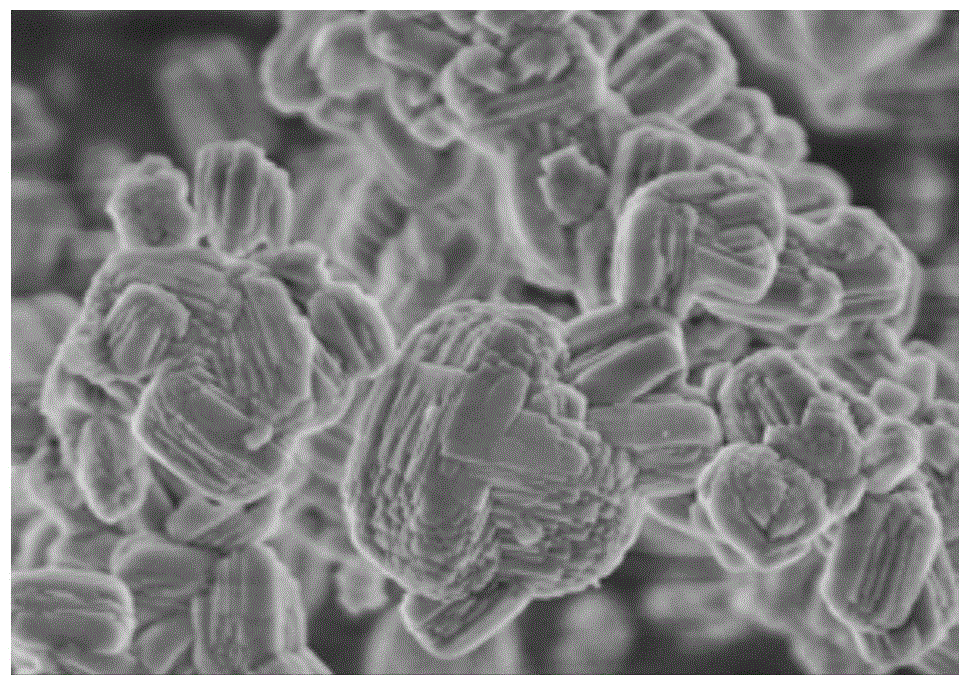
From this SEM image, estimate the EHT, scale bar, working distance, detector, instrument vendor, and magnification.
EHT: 3 kV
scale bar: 3000 nm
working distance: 4.4 mm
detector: SE(U)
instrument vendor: Hitachi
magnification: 18 K X